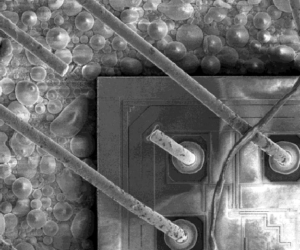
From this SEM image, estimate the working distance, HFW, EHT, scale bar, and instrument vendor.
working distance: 4.6 mm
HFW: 469 µm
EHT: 2 kV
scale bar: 200000 nm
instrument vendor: FEI